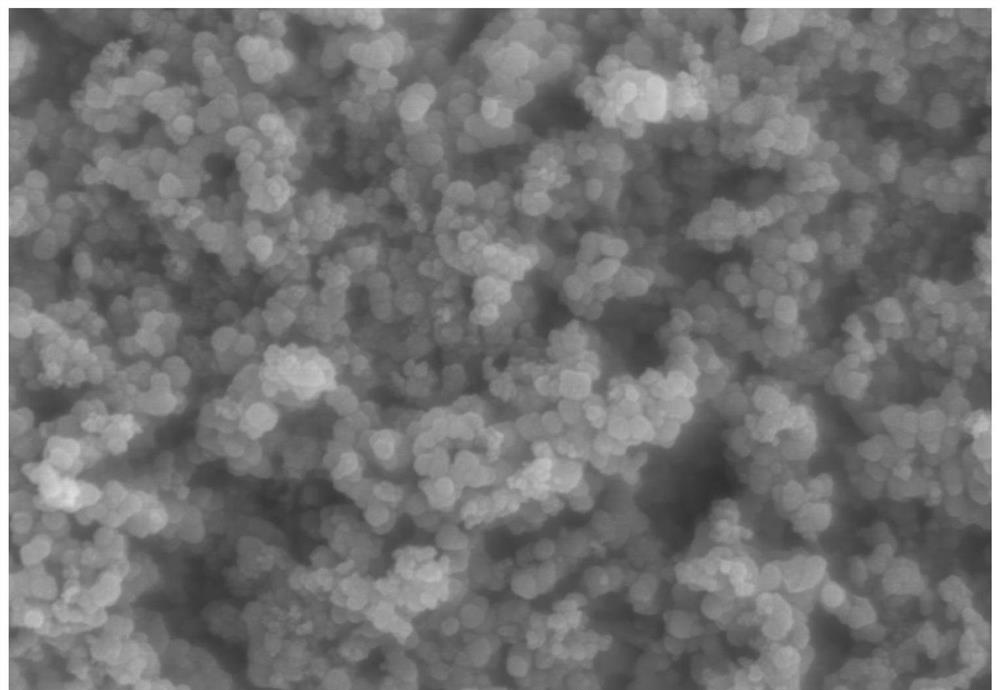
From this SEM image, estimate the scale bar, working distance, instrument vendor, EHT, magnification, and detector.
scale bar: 1000 nm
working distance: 3.3 mm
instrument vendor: Hitachi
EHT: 3 kV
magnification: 50 K X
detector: SE(UL)